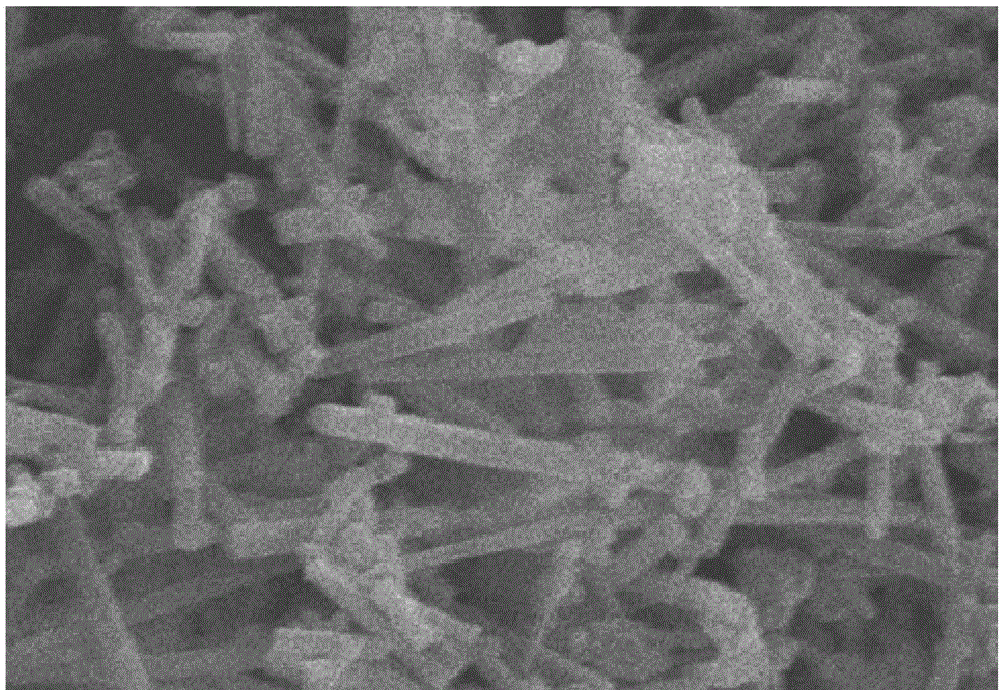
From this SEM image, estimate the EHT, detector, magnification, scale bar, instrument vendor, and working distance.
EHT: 20 kV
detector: SE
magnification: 20 K X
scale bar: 2000 nm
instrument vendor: Hitachi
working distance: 12 mm